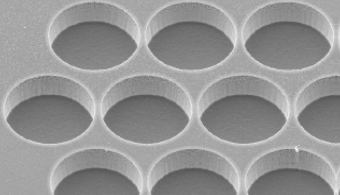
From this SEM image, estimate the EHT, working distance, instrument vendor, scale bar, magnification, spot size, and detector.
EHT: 10 kV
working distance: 10.3 mm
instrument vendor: FEI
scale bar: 50000 nm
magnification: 1.5 K X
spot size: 3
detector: SE